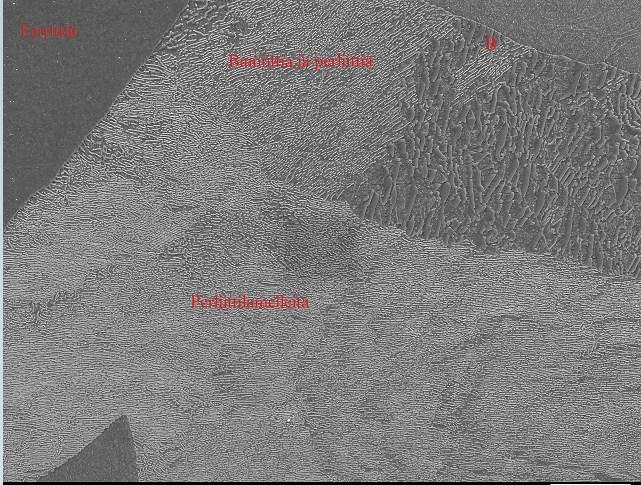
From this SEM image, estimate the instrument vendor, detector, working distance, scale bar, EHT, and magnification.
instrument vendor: JEOL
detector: SEI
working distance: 9.9 mm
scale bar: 10000 nm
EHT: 15 kV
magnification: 1.5 K X